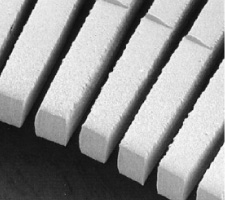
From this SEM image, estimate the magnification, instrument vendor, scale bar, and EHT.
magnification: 0.15 K X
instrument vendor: JEOL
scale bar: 100000 nm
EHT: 10 kV